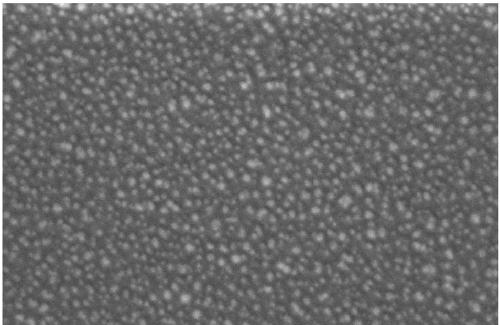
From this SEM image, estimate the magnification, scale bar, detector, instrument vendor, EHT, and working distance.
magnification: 100 K X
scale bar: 100 nm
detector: InLens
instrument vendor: Zeiss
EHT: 5 kV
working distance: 4.9 mm